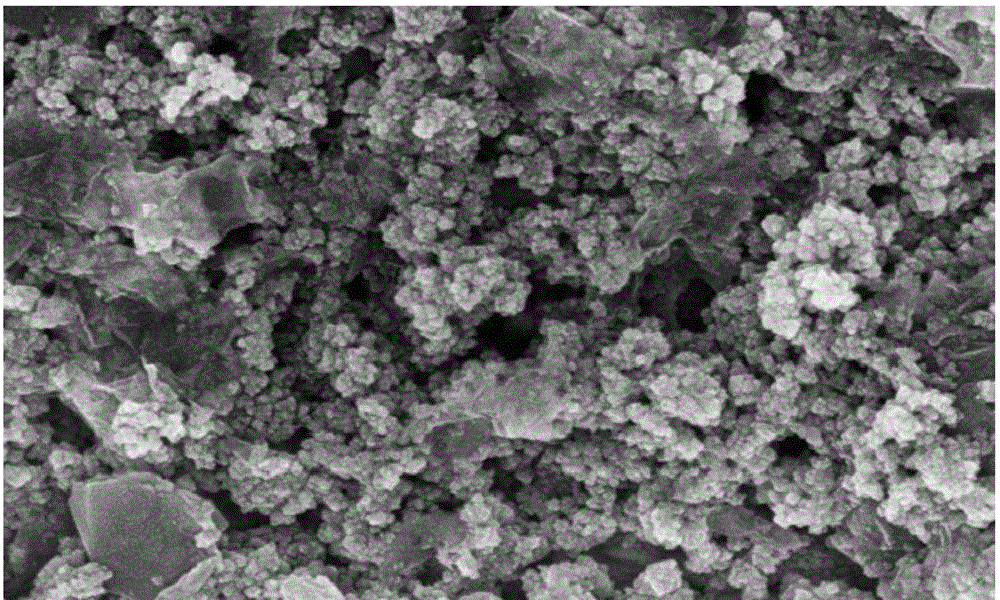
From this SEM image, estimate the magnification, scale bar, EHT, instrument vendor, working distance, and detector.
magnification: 50 K X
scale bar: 200 nm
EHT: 5 kV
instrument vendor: Zeiss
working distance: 6.6 mm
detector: InLens